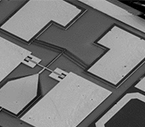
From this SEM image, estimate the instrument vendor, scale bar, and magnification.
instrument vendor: JEOL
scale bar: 100000 nm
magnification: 0.11 K X